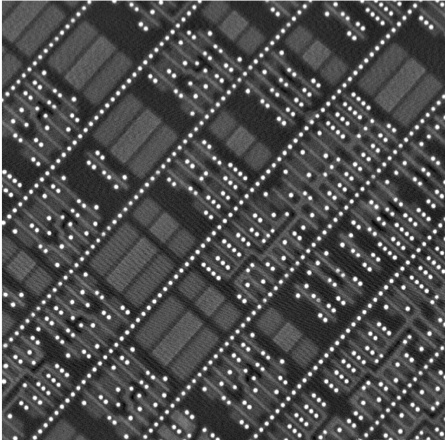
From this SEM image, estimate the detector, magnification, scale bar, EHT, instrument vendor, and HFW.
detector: BSD Full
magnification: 20 K X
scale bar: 3000 nm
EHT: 10 kV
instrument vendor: other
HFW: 13.5 µm